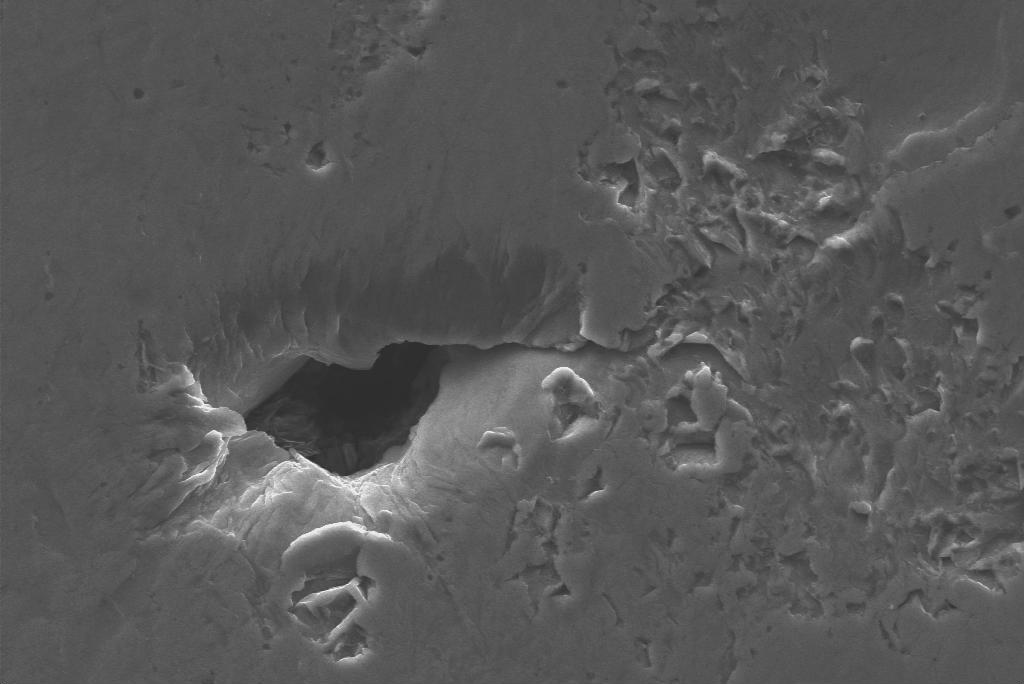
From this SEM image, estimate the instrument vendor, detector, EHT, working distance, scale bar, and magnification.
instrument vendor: Zeiss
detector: SE1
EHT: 20 kV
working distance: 14 mm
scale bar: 10000 nm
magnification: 4 K X